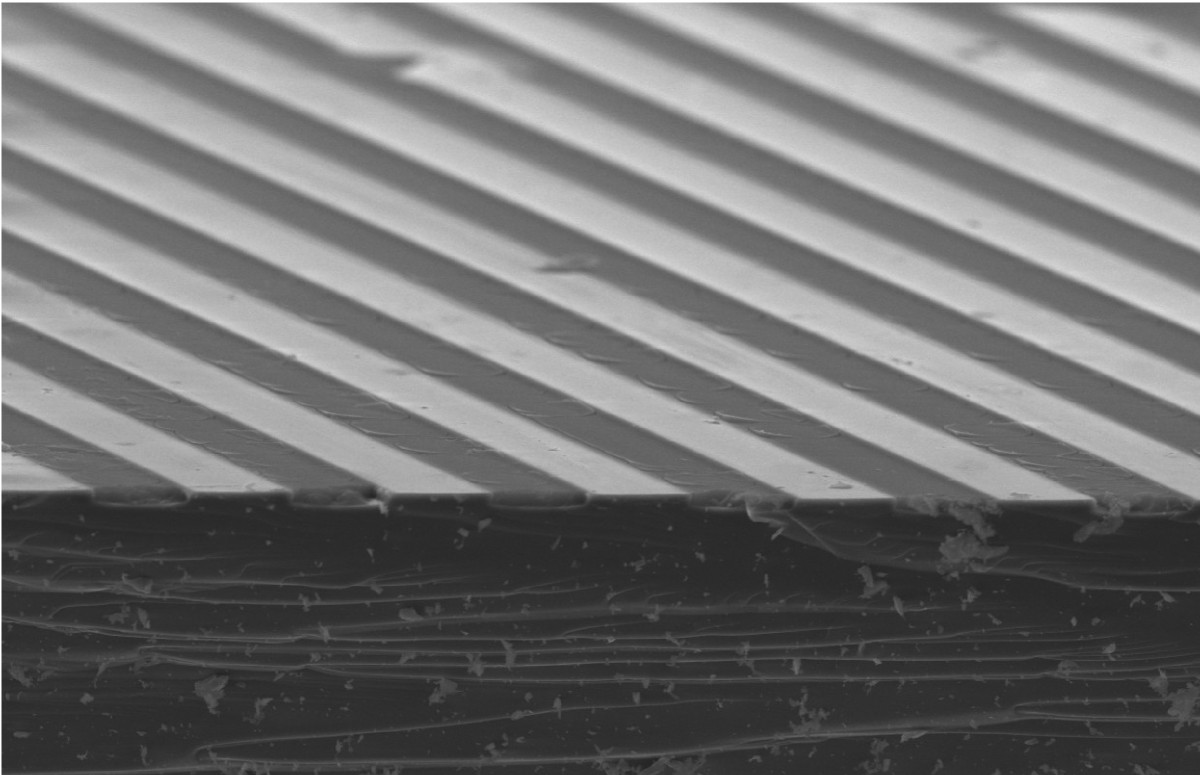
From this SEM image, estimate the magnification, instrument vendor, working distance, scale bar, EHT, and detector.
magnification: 0.3 K X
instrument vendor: Hitachi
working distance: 12.6 mm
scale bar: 100000 nm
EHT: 20 kV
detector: SE